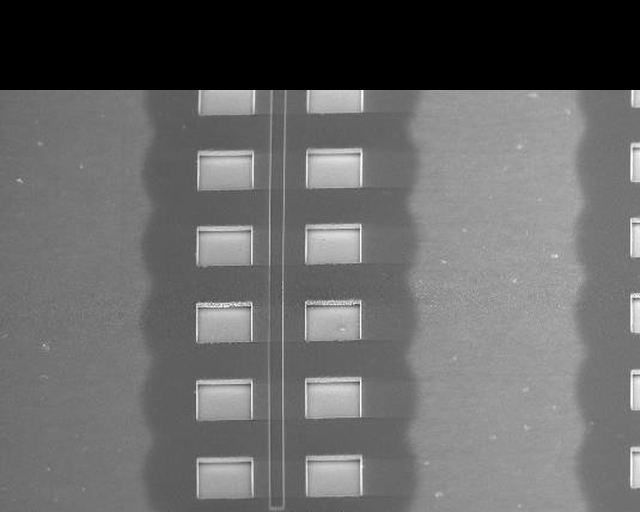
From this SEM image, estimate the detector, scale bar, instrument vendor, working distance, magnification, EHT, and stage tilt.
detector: TLD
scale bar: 40000 nm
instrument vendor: FEI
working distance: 4 mm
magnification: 1.258 K X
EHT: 2 kV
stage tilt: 45°